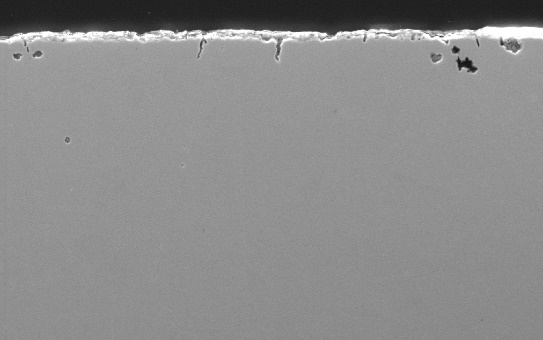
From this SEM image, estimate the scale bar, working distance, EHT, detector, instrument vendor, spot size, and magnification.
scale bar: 50000 nm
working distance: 10 mm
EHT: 20 kV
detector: SE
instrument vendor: FEI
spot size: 4.5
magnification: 0.5 K X